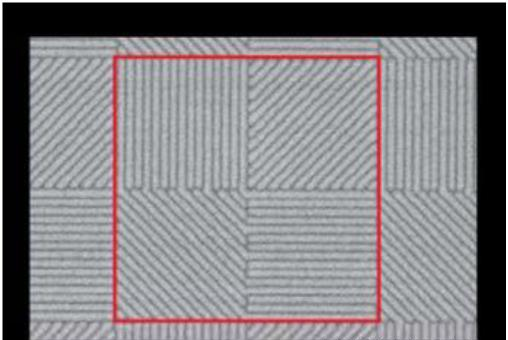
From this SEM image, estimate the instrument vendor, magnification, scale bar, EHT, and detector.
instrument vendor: Hitachi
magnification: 8 K X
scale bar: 5000 nm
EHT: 15 kV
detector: SE(U)